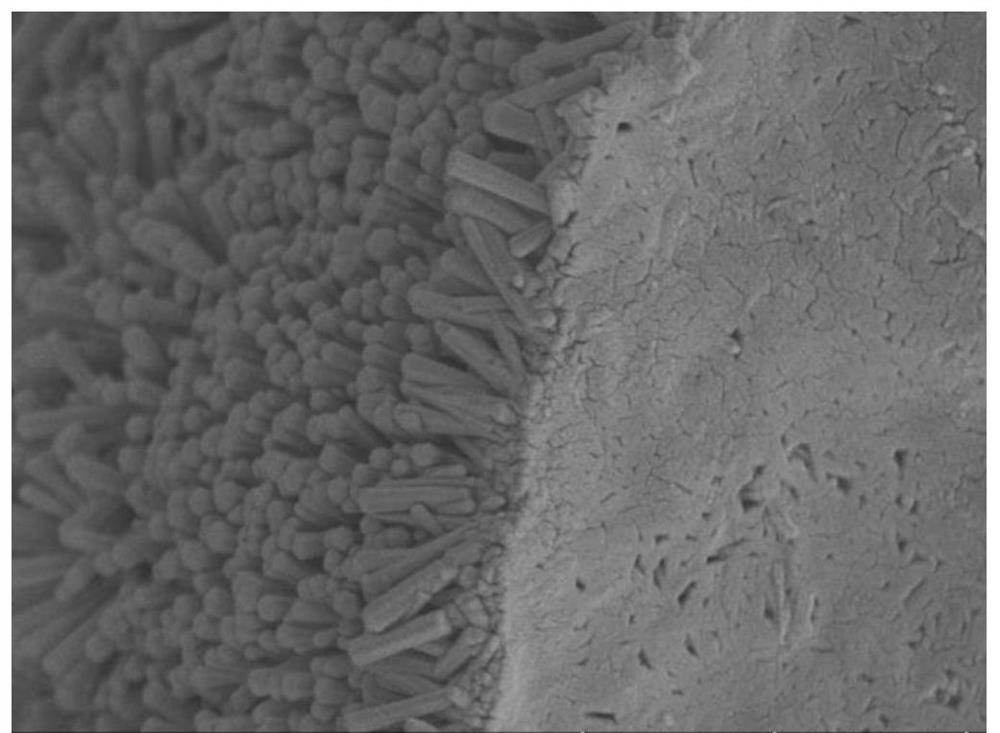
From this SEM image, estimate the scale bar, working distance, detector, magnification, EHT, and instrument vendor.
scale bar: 5000 nm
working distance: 8.5 mm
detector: SE(UL)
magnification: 10 K X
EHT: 5 kV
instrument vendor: Hitachi